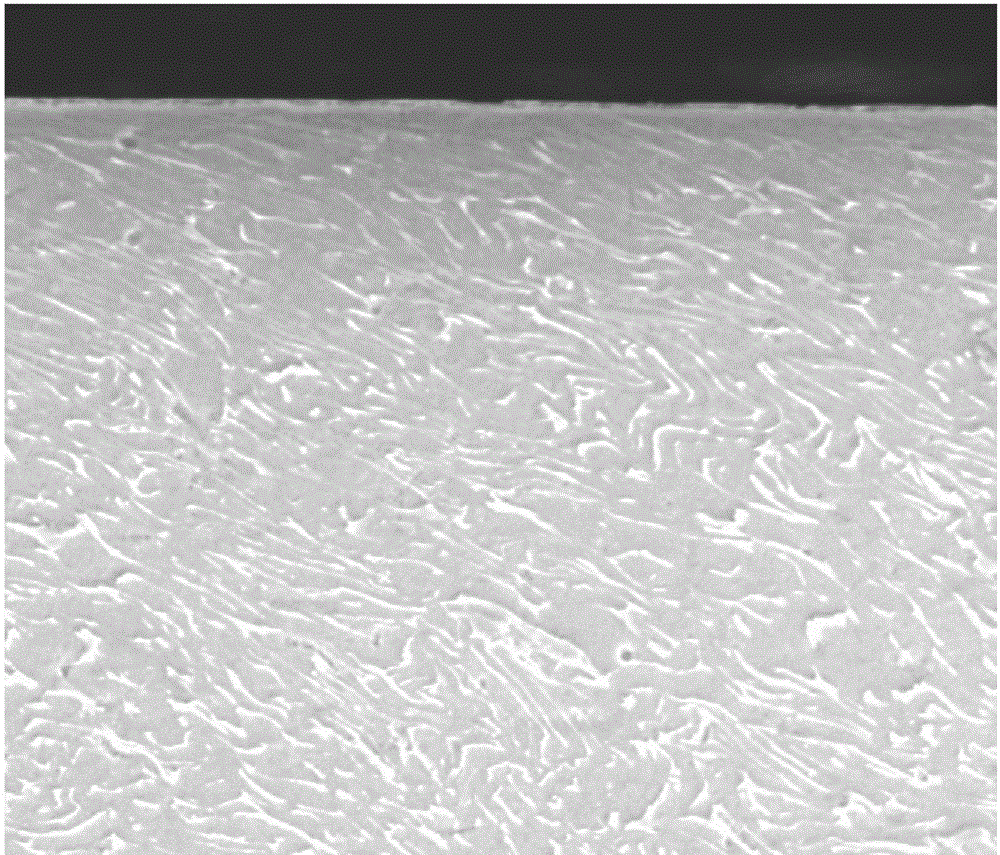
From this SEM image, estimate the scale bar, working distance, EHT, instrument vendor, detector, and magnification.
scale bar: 40000 nm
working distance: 11.8 mm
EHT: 15 kV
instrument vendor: FEI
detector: ETD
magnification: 3 K X